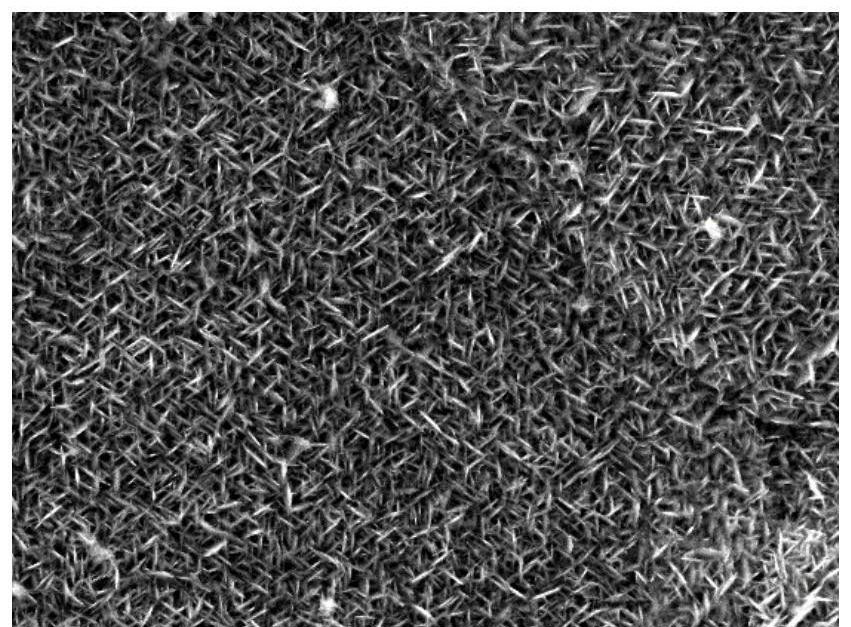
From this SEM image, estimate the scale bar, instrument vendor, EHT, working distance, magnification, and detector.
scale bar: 1000 nm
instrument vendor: JEOL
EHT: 10 kV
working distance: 9.9 mm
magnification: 10 K X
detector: SED